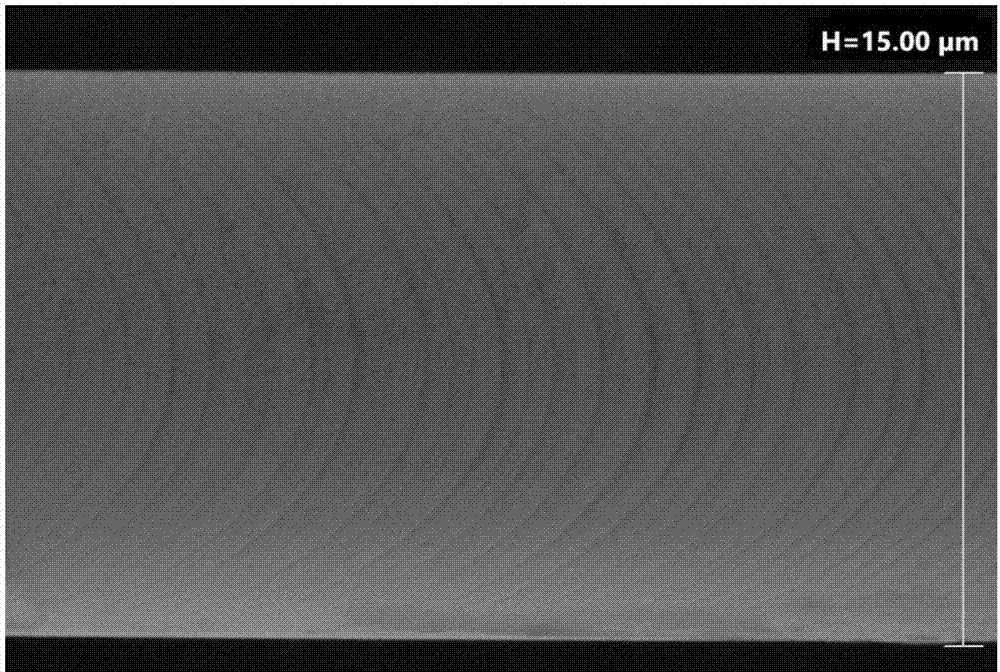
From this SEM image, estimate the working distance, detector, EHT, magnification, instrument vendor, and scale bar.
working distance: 11.2 mm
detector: SE2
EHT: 20 kV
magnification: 11.56 K X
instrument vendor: Zeiss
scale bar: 2000 nm